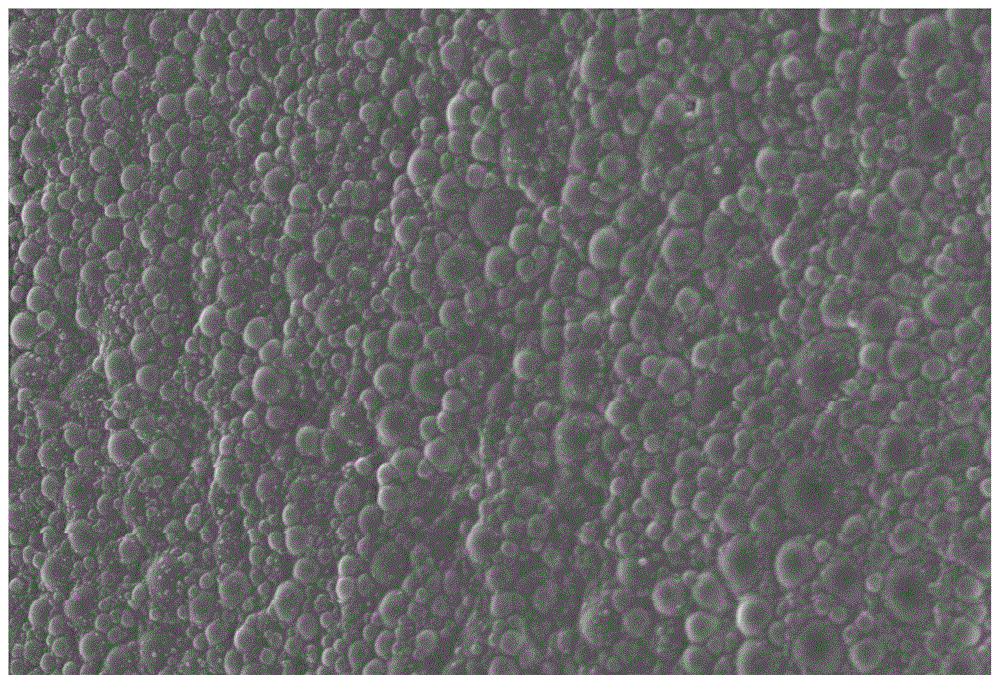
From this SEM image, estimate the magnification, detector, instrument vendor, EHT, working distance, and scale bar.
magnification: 9.49 K X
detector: InLens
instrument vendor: Zeiss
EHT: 5 kV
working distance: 7.4 mm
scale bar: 2000 nm